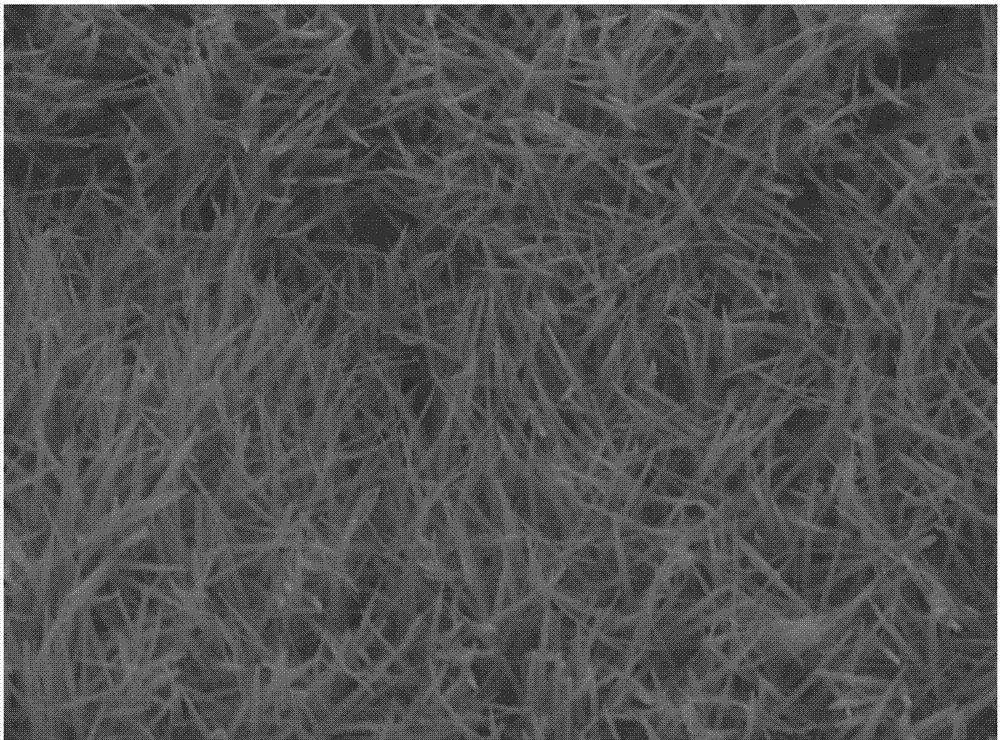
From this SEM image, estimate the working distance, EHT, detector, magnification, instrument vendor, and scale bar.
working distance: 10.1 mm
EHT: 10 kV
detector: LED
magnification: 10 K X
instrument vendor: JEOL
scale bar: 1000 nm